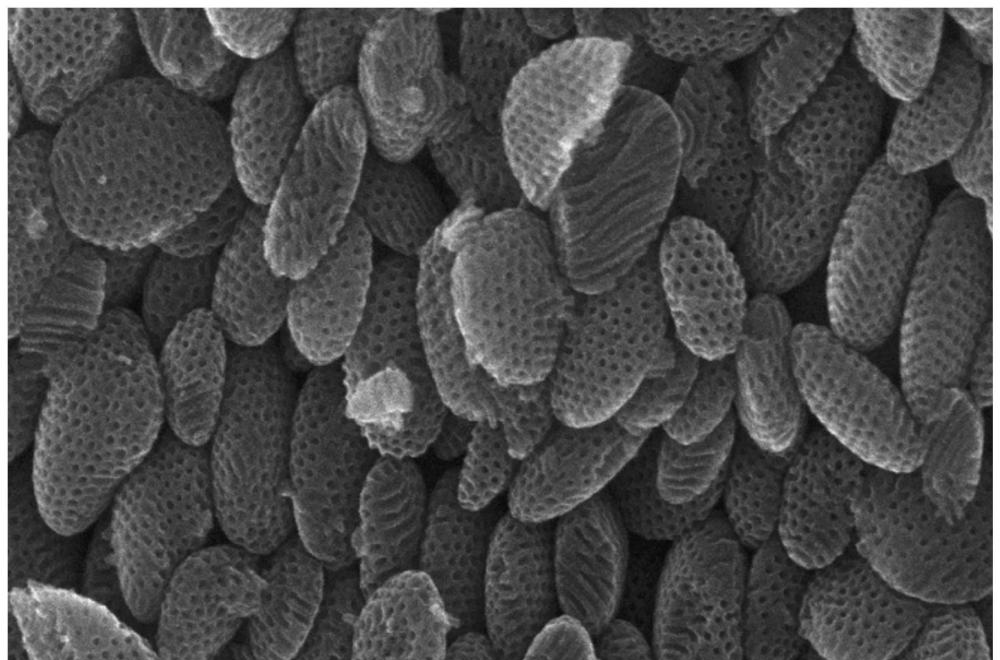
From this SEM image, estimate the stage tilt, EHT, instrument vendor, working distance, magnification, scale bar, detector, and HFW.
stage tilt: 0°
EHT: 10 kV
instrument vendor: FEI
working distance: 4.6 mm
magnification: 65 K X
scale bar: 500 nm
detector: TLD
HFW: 1.95 µm